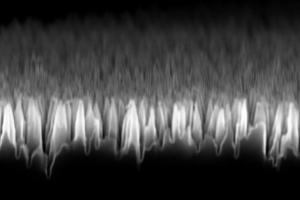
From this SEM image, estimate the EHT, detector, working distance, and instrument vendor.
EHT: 10 kV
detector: InLens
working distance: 5 mm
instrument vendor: Zeiss